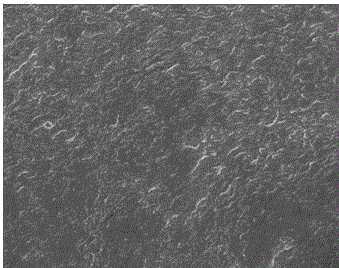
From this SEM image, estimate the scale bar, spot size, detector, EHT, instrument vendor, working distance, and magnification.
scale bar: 3000 nm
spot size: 3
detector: TLD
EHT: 10 kV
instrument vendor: FEI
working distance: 5.5 mm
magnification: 20 K X